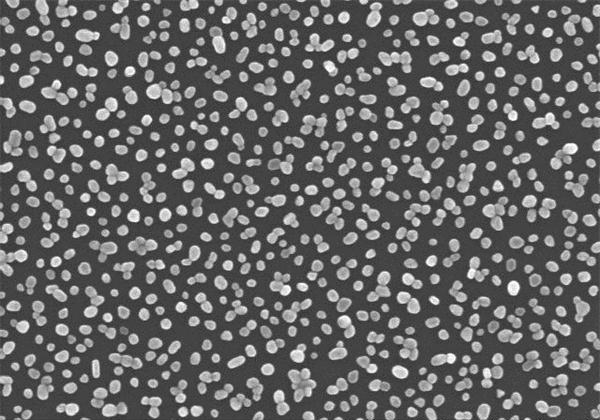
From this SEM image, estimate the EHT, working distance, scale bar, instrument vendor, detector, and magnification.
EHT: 15 kV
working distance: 8.5 mm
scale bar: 1000 nm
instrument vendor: Hitachi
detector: SE(U)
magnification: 50 K X